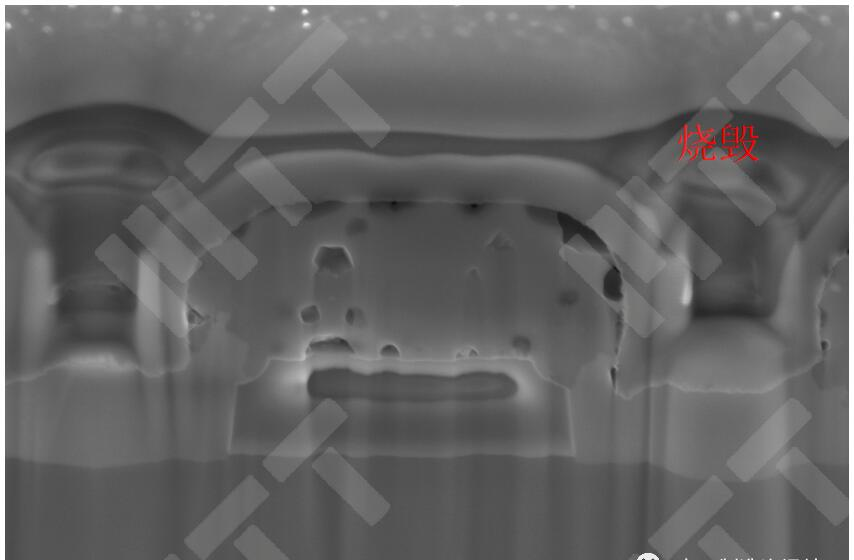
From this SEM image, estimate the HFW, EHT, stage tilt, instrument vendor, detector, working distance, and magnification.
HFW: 5.08 µm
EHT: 5 kV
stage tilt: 52°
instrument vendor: FEI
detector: TLD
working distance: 4 mm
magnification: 25 K X